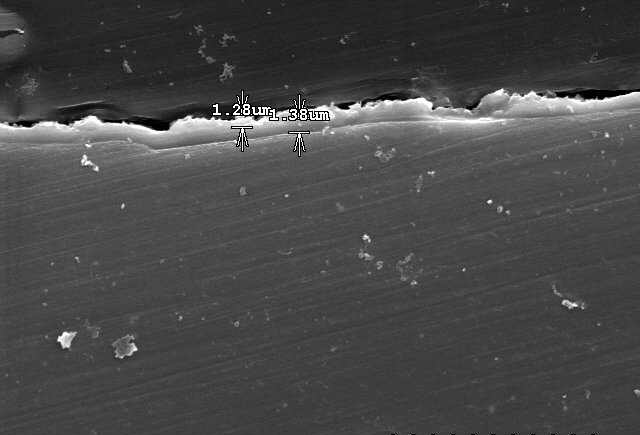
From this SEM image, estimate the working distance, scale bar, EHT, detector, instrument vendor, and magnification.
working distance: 13.6 mm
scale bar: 20000 nm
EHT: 15 kV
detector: SE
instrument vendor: Hitachi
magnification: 2 K X